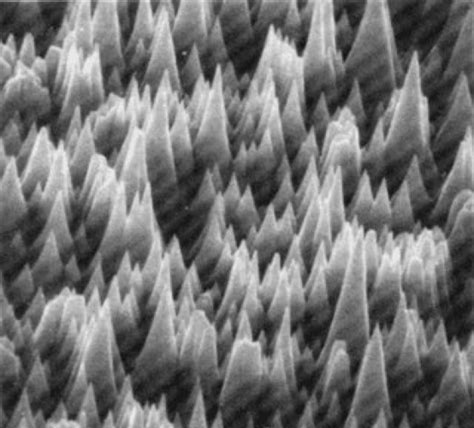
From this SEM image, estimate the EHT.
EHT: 30 kV